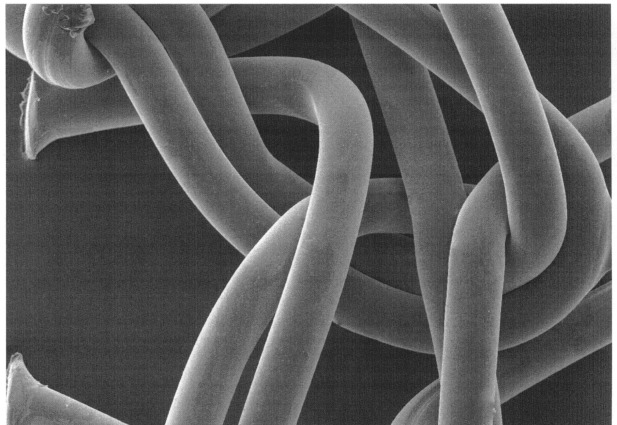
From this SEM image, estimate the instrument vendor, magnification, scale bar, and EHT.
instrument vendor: JEOL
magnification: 0.1 K X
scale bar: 100000 nm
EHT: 15 kV